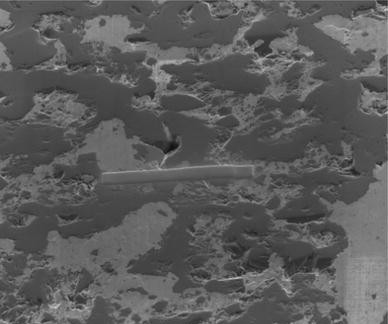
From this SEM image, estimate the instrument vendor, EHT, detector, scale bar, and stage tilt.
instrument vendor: FEI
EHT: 5 kV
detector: ETD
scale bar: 20000 nm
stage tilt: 52°